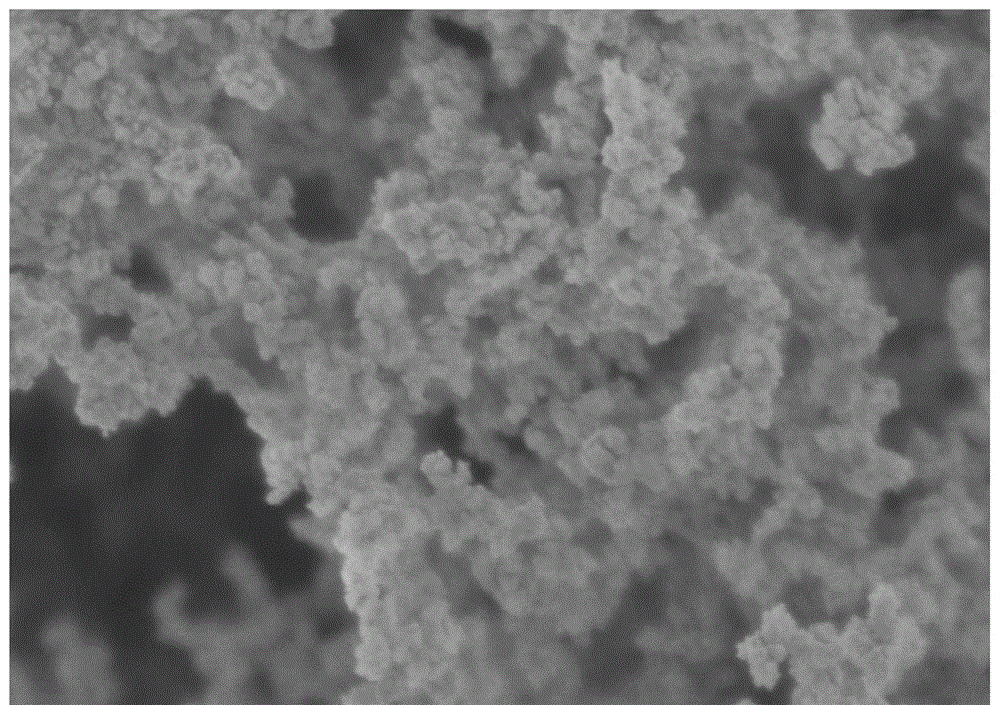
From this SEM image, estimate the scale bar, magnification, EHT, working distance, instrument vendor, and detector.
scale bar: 500 nm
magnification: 100 K X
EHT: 1 kV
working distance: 3 mm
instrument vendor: Hitachi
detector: SE(U)